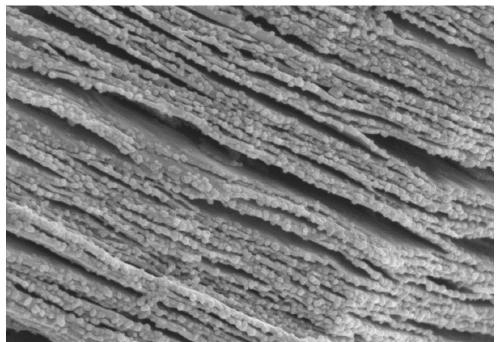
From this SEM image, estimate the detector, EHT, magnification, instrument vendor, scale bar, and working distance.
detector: SE(M)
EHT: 3 kV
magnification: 50 K X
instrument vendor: Hitachi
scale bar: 1000 nm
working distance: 9.2 mm